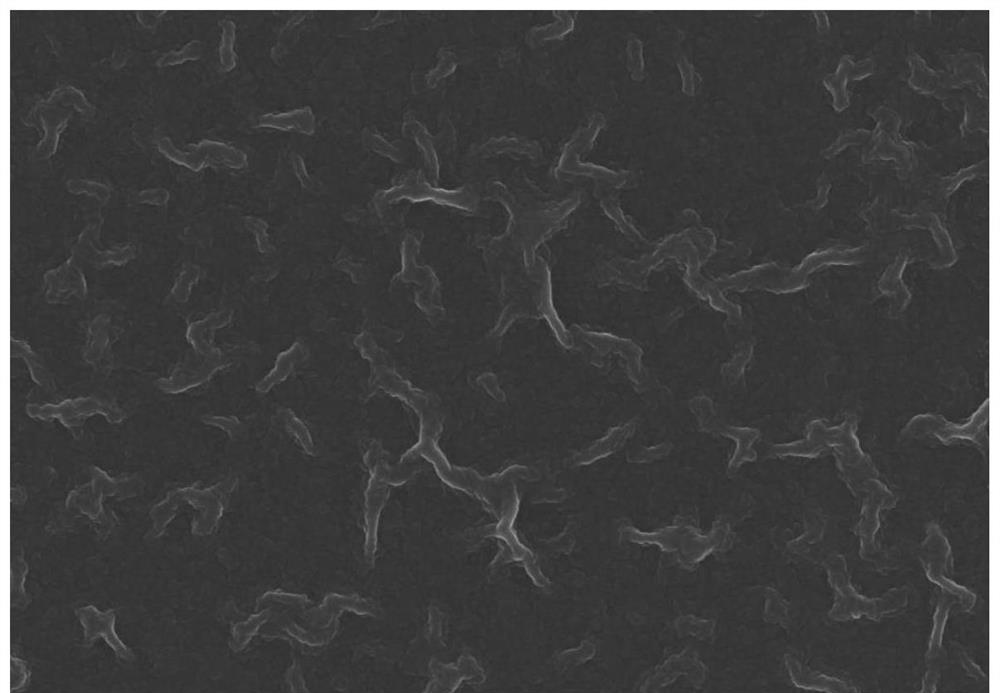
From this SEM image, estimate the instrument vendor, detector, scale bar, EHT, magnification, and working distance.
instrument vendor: Zeiss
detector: InLens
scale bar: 1000 nm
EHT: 5 kV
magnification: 20 K X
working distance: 4.7 mm